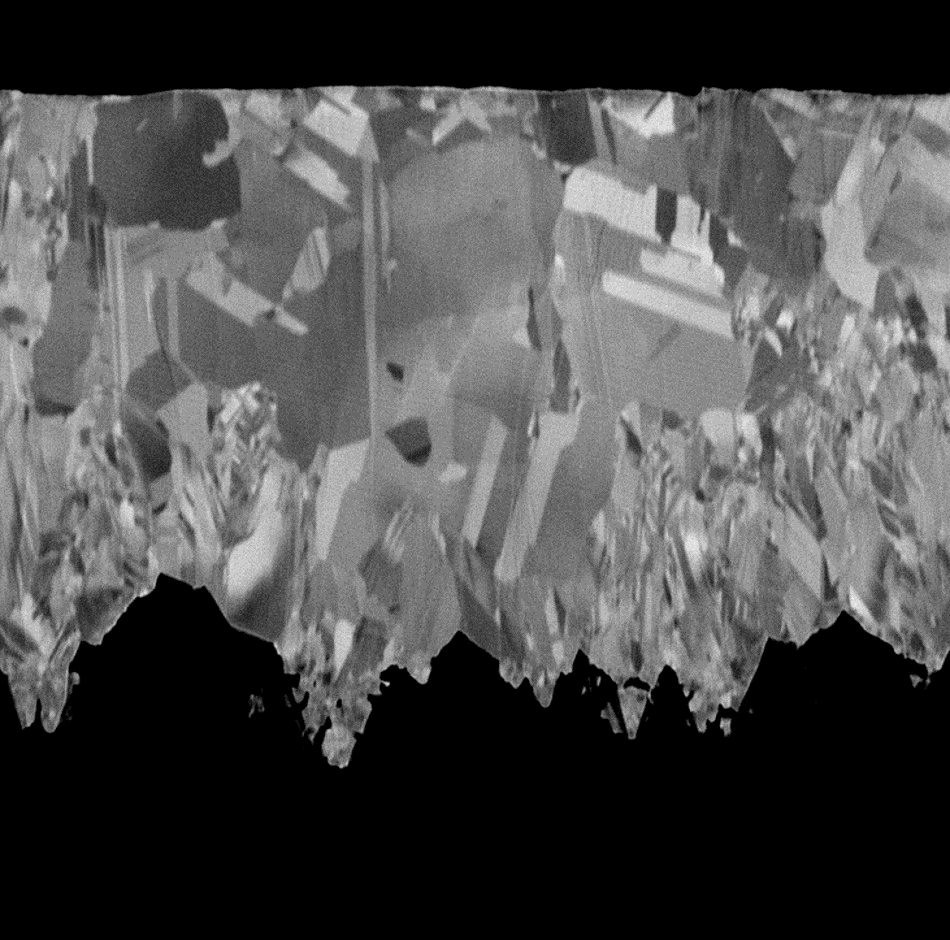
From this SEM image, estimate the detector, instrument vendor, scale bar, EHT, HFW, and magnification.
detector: BSD Full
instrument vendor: other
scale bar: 10000 nm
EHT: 5 kV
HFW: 54.2 µm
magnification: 5 K X